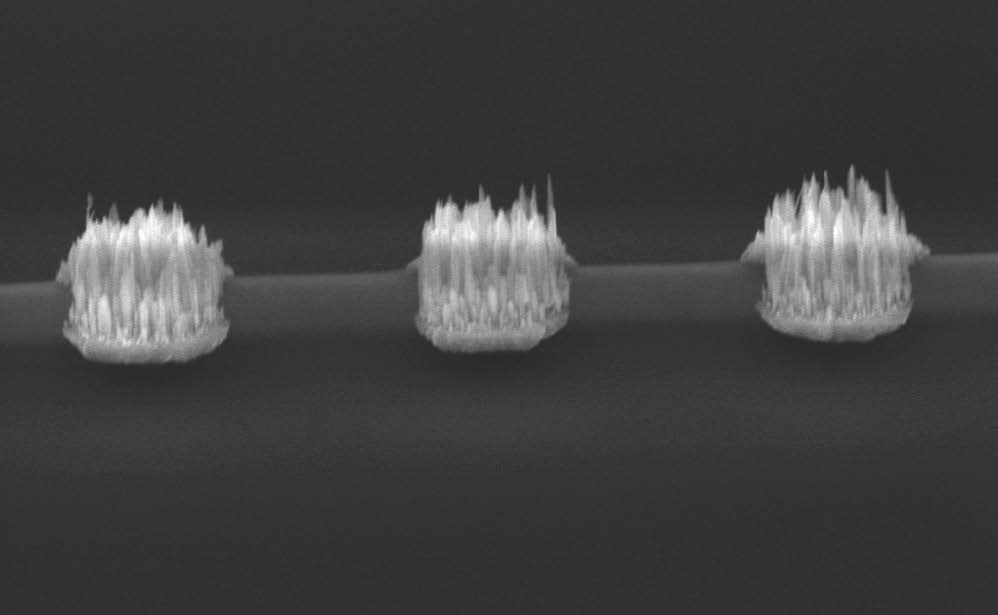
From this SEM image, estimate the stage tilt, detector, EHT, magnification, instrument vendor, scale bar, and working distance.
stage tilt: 62.8°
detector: SE1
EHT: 28 kV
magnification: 5.38 K X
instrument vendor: Zeiss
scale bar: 4000 nm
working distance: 24.66 mm